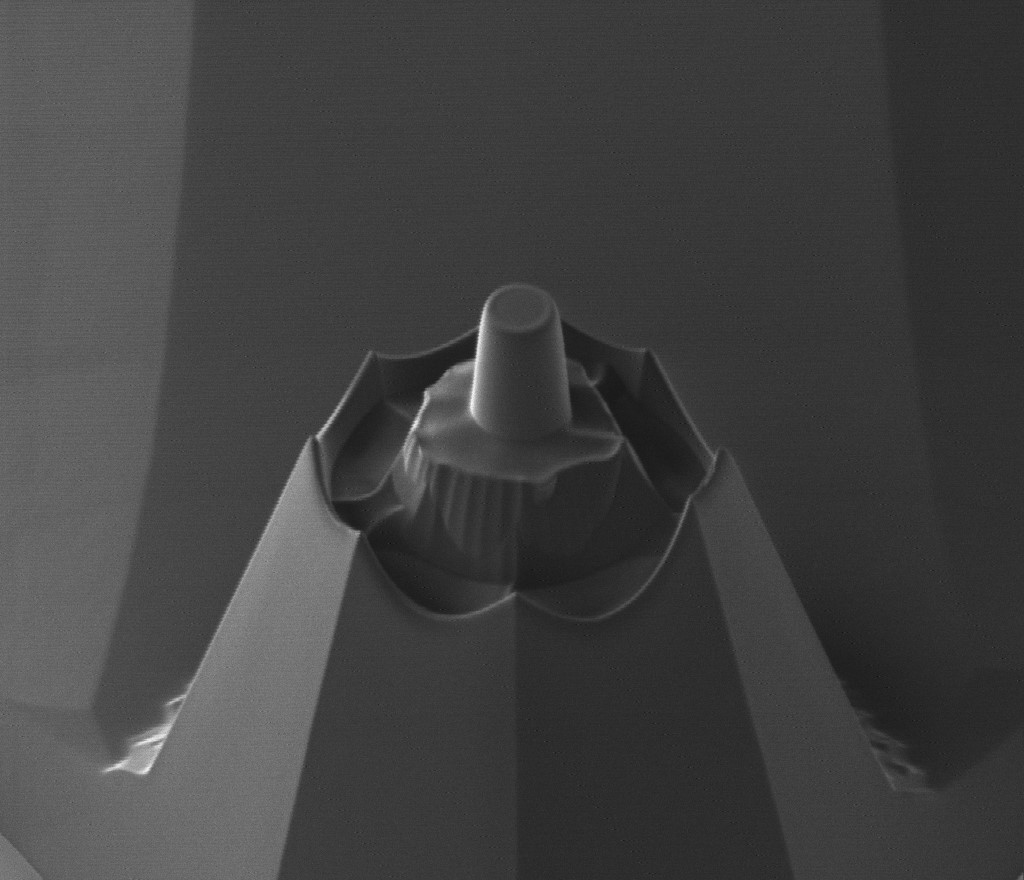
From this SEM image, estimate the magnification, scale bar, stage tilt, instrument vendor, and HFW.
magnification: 12 K X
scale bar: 5000 nm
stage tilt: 45°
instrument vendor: FEI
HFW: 25.3 µm